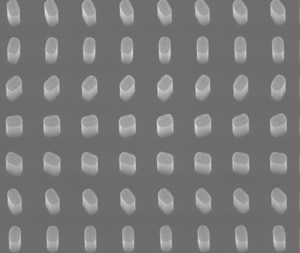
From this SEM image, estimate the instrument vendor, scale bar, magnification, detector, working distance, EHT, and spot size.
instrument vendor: FEI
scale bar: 1000 nm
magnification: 136.256 K X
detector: ETD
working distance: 10.48 mm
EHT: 20 kV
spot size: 4